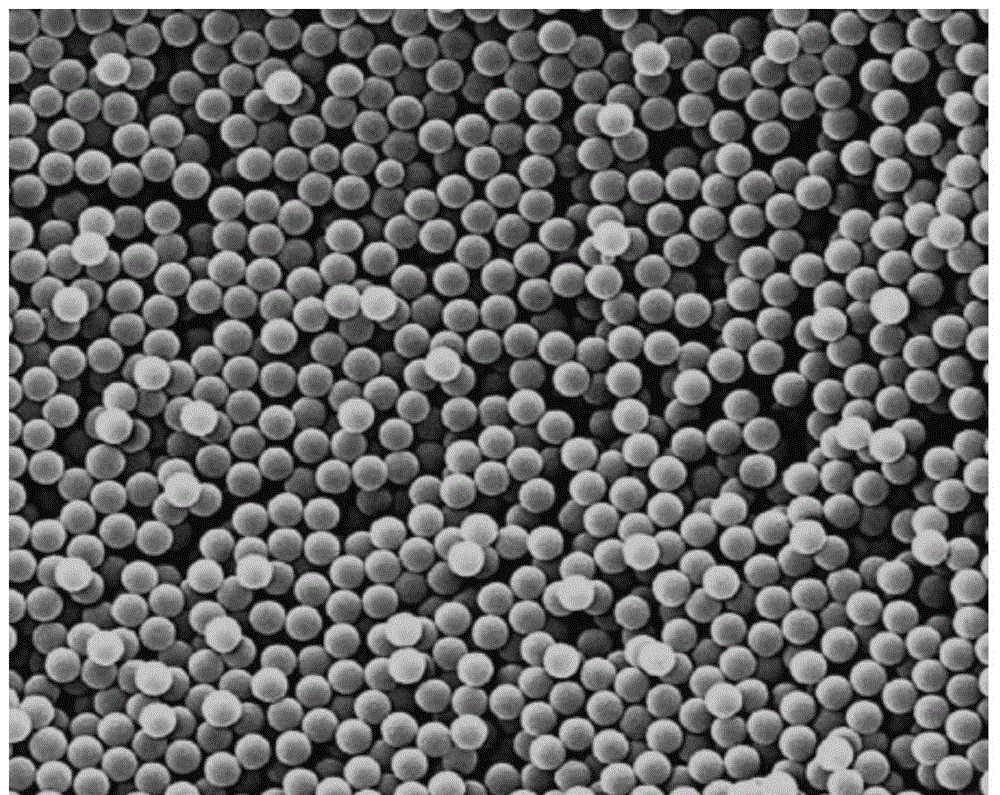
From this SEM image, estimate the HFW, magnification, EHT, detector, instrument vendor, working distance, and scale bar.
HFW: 12.8 µm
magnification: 20 K X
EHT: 20 kV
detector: ETD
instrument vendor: FEI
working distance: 11.4 mm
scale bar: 5000 nm